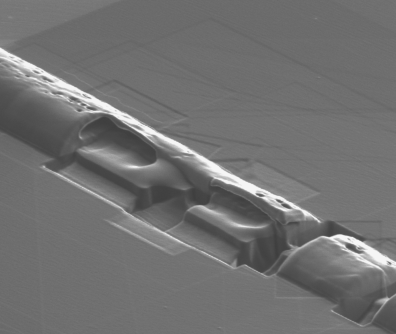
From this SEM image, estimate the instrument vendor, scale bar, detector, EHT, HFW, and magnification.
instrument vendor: FEI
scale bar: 4000 nm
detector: ETD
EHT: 30 kV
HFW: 9.69 µm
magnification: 27.91 K X